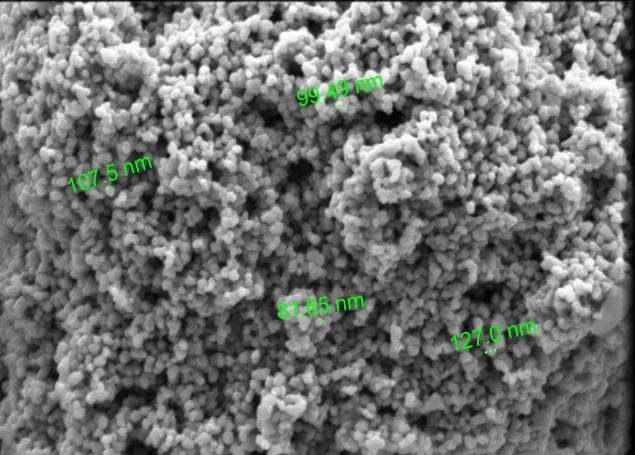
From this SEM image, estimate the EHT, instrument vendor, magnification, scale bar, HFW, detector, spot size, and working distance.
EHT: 5 kV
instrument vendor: FEI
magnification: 50 K X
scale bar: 2000 nm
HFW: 8.29 µm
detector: ETD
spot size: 3.5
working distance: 9.9 mm